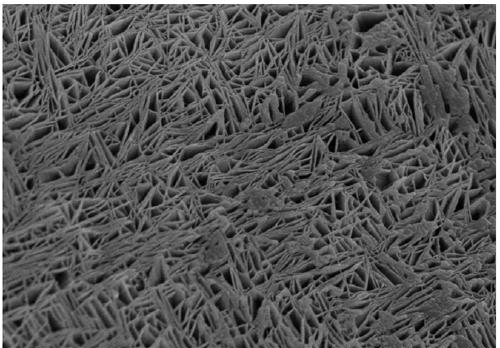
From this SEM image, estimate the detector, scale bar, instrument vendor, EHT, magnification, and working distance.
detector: SE(M)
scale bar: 2000 nm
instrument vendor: Hitachi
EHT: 5 kV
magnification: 20 K X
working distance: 9.4 mm